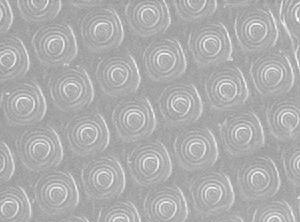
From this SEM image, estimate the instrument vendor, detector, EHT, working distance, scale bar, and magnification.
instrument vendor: JEOL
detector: SEI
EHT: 3 kV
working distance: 15 mm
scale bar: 1000 nm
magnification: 3 K X